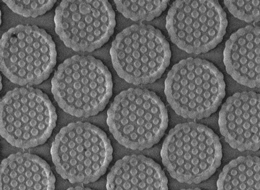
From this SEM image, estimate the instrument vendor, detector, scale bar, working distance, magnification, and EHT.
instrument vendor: JEOL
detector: LED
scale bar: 1000 nm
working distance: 9 mm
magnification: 10 K X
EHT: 1.5 kV